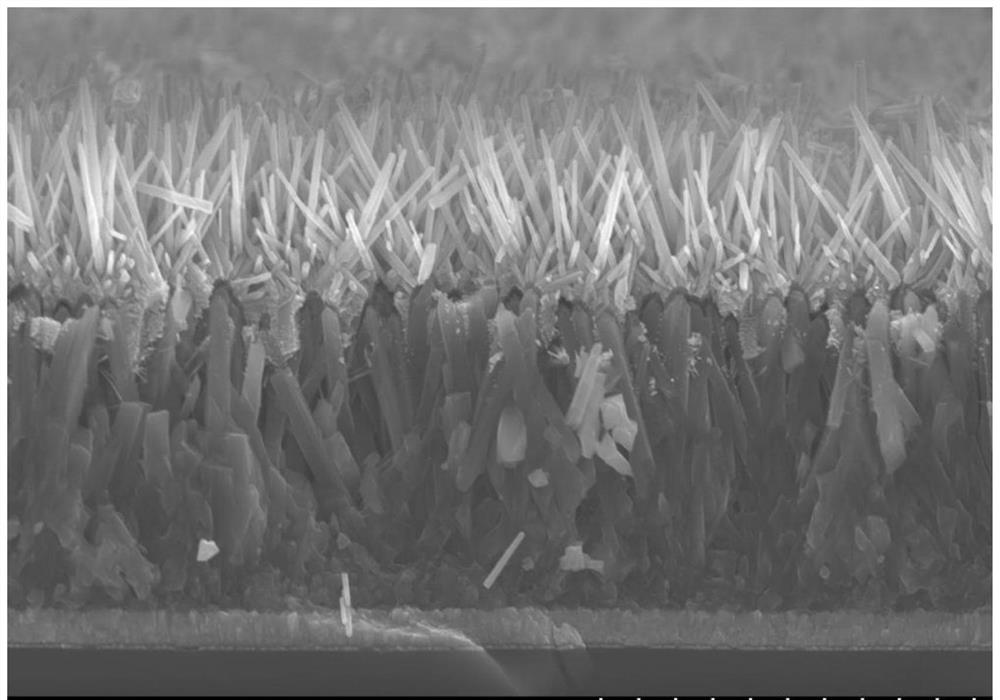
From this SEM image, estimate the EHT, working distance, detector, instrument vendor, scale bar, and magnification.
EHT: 10 kV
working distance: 10.5 mm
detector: SE(M)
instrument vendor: Hitachi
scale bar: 4000 nm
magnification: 12 K X